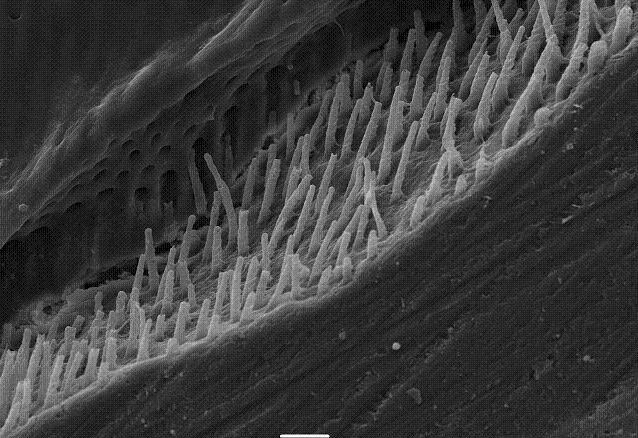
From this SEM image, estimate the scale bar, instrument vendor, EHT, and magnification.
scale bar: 10000 nm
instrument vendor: JEOL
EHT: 15 kV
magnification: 1 K X